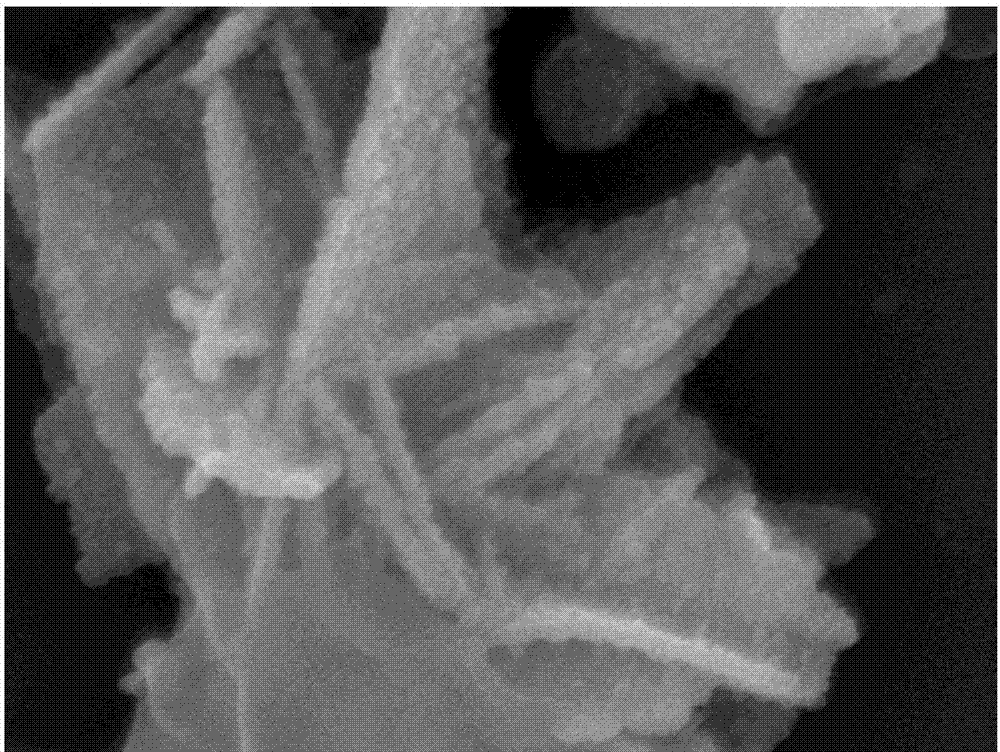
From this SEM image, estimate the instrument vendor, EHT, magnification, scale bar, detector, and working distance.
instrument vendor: JEOL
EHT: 8 kV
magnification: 100 K X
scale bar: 100 nm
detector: SEI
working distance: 8.1 mm